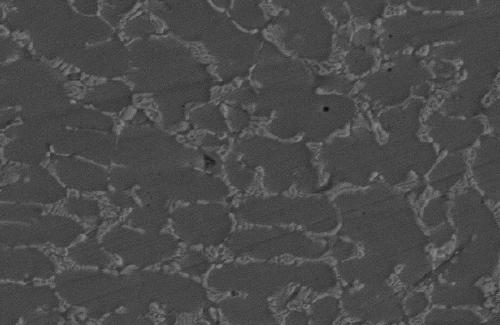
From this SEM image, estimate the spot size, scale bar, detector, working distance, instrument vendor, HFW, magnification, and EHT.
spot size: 6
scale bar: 10000 nm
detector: TLD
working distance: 12.4 mm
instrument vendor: FEI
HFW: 41.4 µm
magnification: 5 K X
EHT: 20 kV